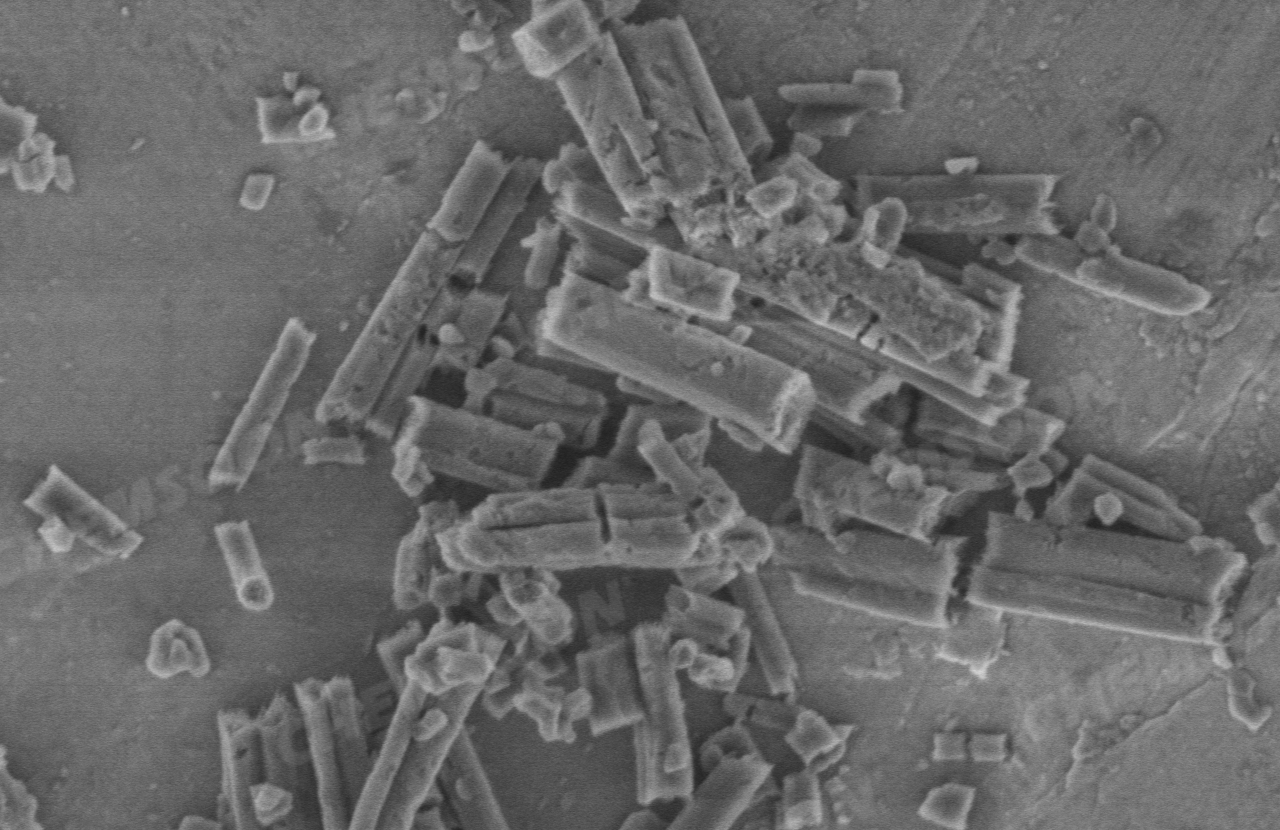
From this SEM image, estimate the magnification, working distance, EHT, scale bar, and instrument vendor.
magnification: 40 K X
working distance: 2.5 mm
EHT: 1 kV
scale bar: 1000 nm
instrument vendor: Hitachi